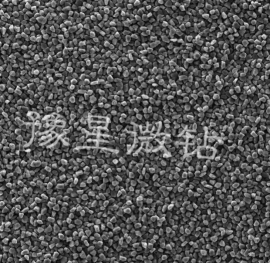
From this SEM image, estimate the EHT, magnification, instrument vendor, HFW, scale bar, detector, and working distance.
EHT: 20 kV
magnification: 1 K X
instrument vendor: Tescan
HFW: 225 µm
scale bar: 50000 nm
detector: SE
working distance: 9.98 mm